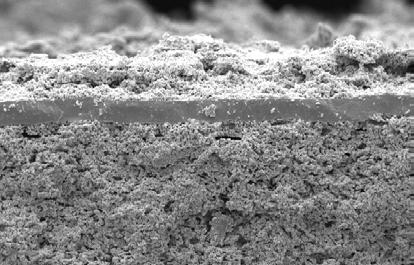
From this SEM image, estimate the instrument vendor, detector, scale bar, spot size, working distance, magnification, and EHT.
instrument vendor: FEI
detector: SE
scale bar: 50000 nm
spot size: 3.3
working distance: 16.1 mm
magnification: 0.5 K X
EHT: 15 kV